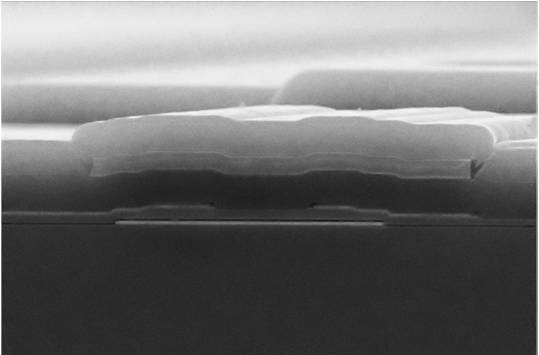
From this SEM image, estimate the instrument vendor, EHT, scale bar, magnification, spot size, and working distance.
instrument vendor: other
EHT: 20 kV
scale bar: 3000 nm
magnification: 10 K X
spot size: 8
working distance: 5 mm